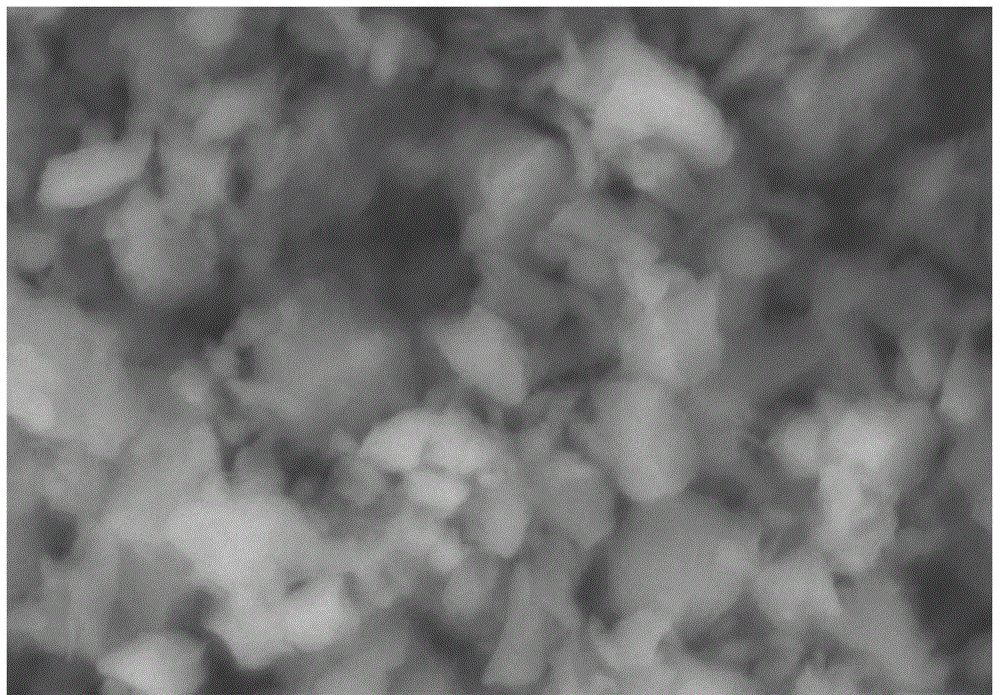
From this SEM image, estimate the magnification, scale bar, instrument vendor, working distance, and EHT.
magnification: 30 K X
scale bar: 1000 nm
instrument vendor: Hitachi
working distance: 9.6 mm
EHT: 10 kV